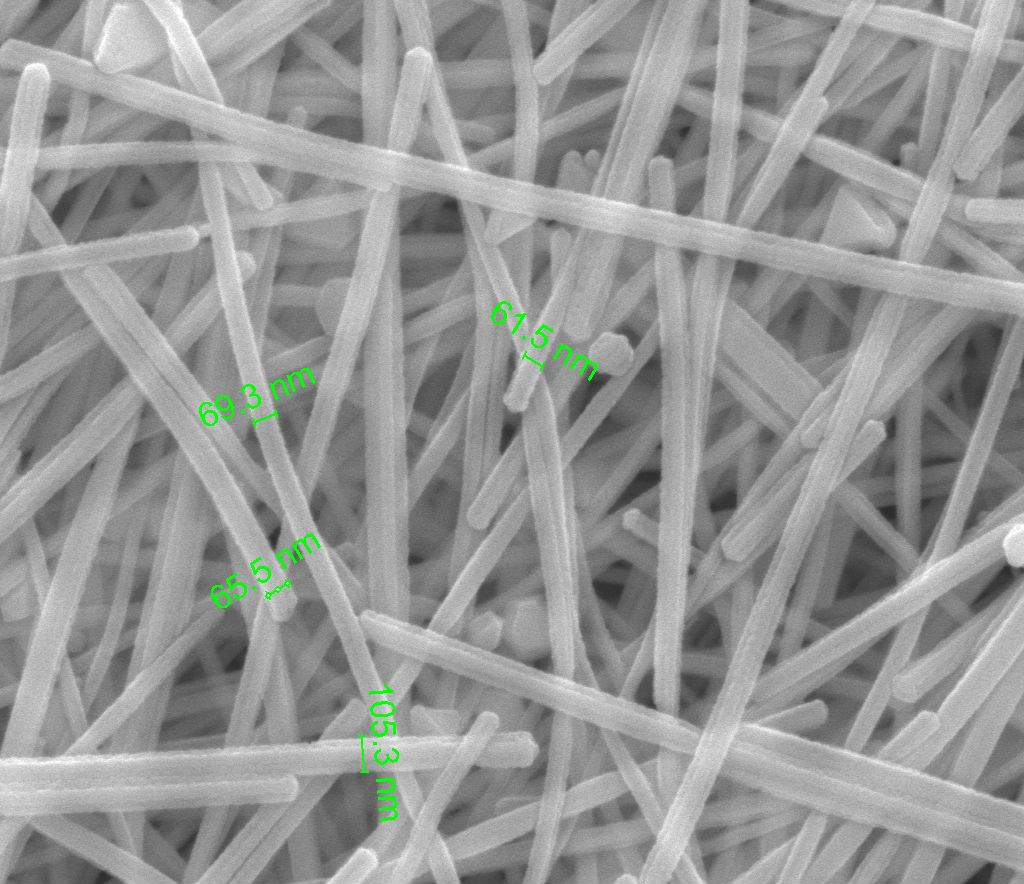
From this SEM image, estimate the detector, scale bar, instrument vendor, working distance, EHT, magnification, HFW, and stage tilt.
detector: ETD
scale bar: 1000 nm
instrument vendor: FEI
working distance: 9.9 mm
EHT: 20 kV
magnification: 100 K X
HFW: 2.98 µm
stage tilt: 0°